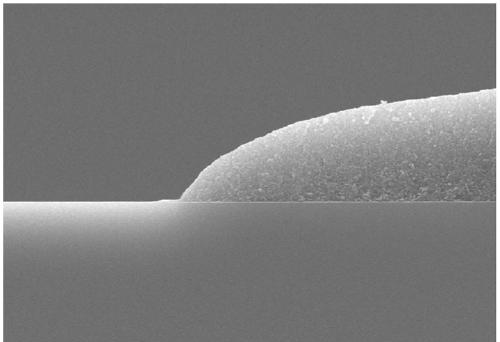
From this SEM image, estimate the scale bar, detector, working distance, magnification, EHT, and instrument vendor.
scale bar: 2000 nm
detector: SE(UL)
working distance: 7.6 mm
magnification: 20 K X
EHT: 10 kV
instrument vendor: Hitachi